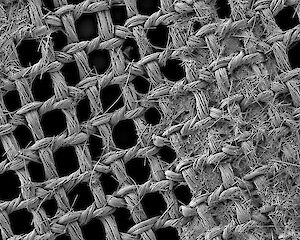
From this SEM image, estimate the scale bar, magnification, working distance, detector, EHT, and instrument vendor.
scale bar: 100000 nm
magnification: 0.033 K X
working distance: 12 mm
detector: LEI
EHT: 3 kV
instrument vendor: JEOL